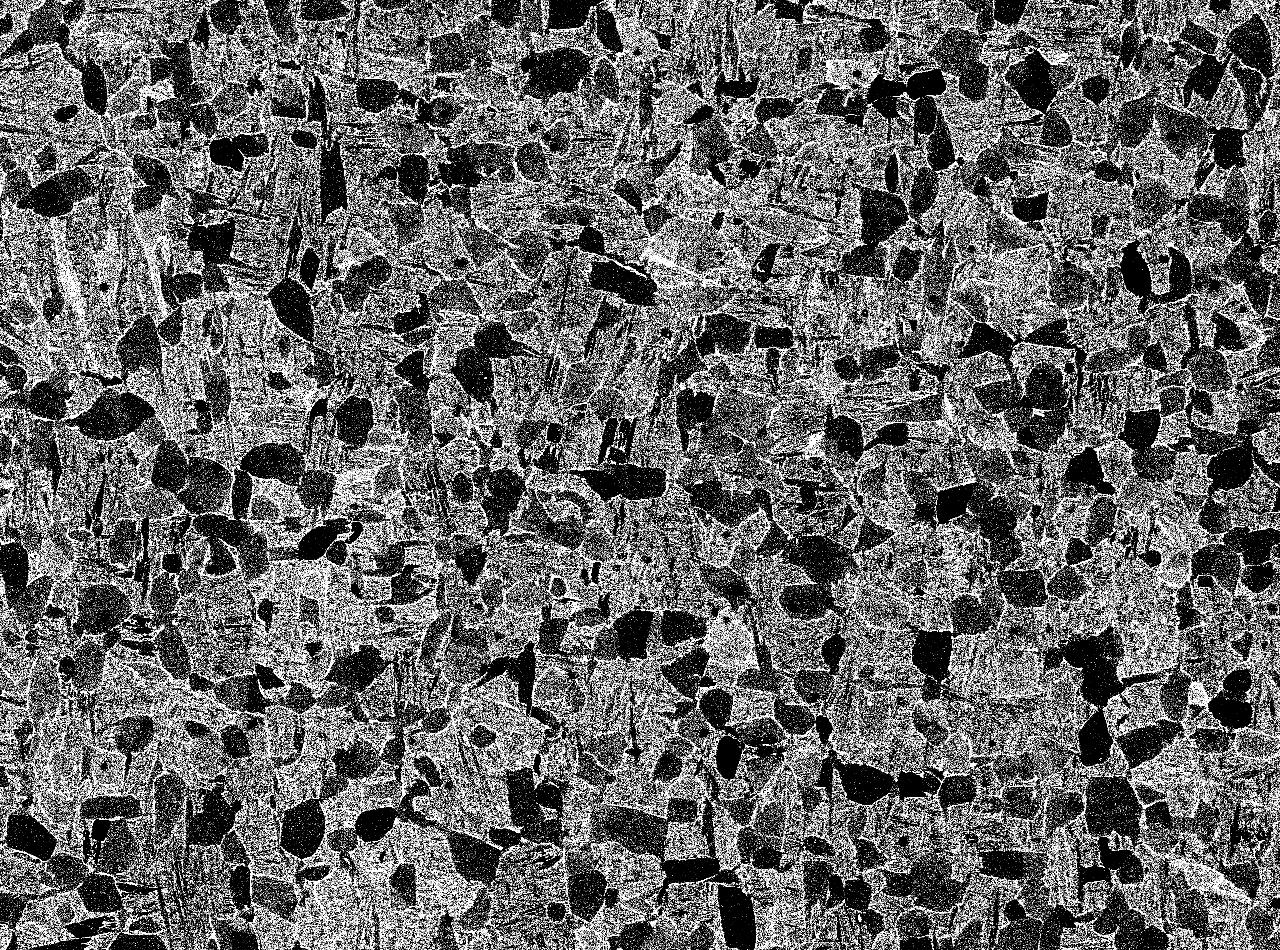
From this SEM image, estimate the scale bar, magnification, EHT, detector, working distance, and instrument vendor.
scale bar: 10000 nm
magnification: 0.5 K X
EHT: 15 kV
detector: COMPO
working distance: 10 mm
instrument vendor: JEOL